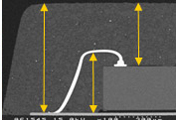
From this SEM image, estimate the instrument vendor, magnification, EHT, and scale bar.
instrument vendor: JEOL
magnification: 0.1 K X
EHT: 15 kV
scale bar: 300000 nm